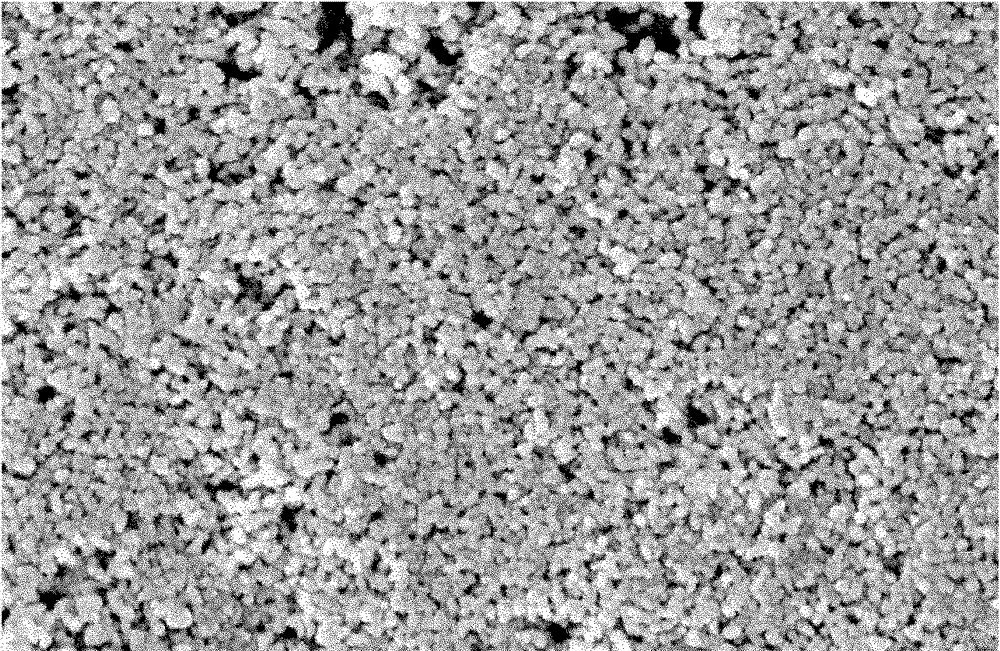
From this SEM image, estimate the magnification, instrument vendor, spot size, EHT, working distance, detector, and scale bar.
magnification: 7.999 K X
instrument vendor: FEI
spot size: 2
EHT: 15 kV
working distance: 10.2 mm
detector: SE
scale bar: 2000 nm